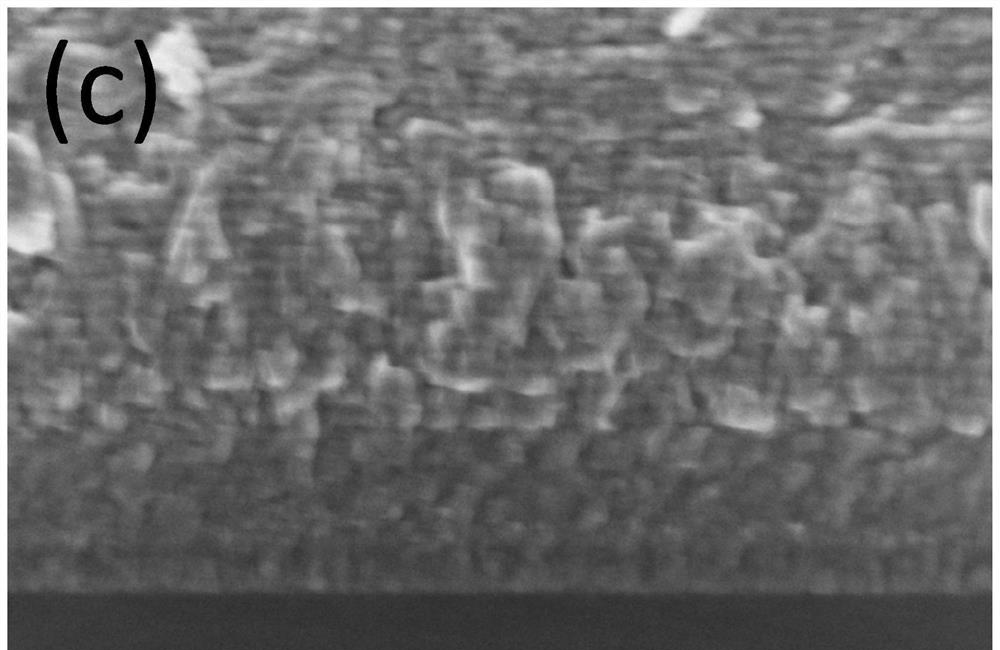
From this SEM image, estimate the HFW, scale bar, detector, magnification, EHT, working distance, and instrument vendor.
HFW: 1.27 µm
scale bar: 200 nm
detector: TLD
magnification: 100 K X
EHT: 2 kV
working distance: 3.7 mm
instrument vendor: Thermo